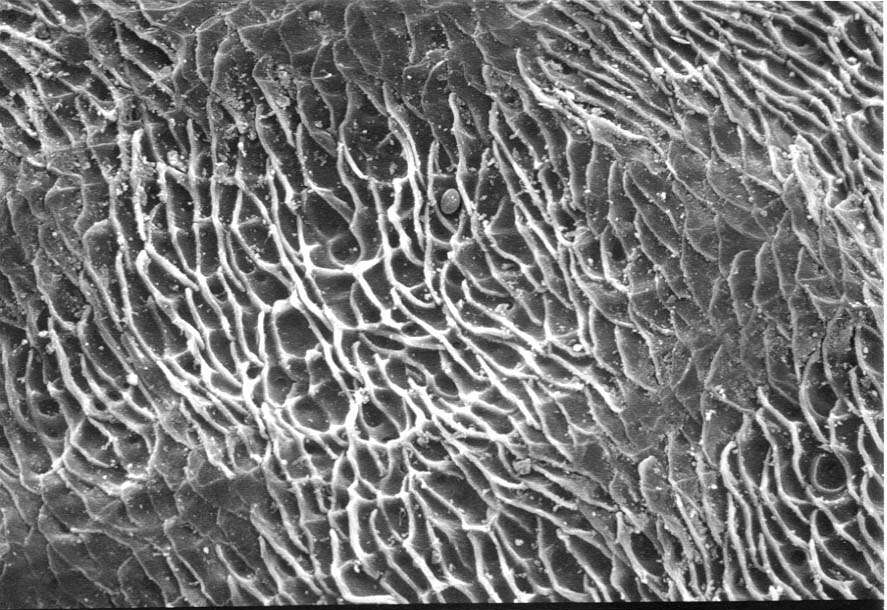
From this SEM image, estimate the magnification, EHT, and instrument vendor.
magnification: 0.5 K X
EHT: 20 kV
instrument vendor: JEOL